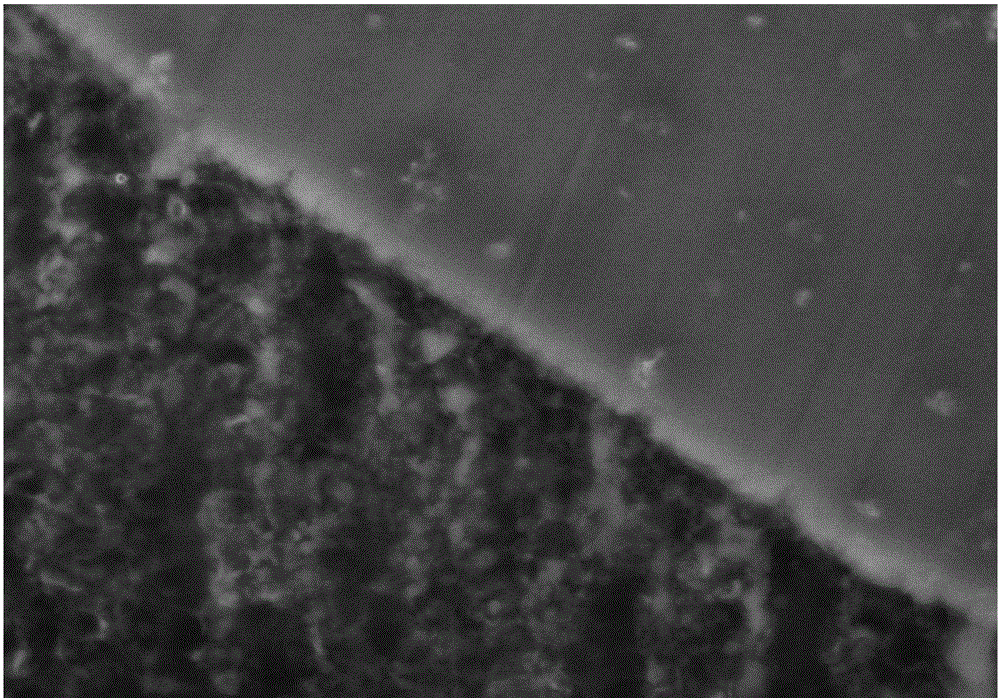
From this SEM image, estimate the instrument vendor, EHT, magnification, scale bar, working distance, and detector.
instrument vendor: Hitachi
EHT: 15 kV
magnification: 30 K X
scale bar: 1000 nm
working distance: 12.6 mm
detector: SE(M,LA3)